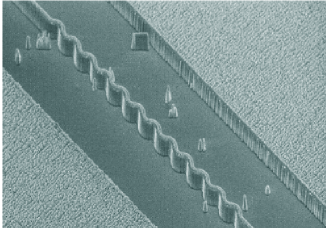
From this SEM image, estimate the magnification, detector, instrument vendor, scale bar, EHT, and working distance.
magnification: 1.81 K X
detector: SE(M)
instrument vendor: Hitachi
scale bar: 30000 nm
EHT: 5 kV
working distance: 12.2 mm